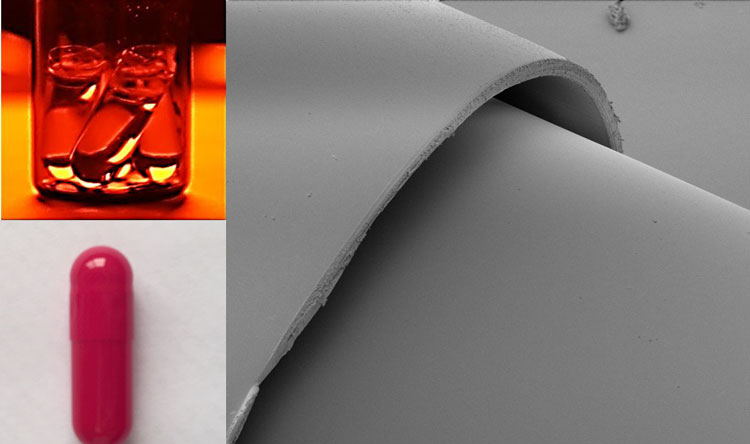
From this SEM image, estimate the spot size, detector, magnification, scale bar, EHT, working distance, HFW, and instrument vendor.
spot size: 2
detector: ETD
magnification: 0.1 K X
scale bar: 500000 nm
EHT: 5 kV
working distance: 52.7 mm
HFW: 2980 µm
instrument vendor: FEI